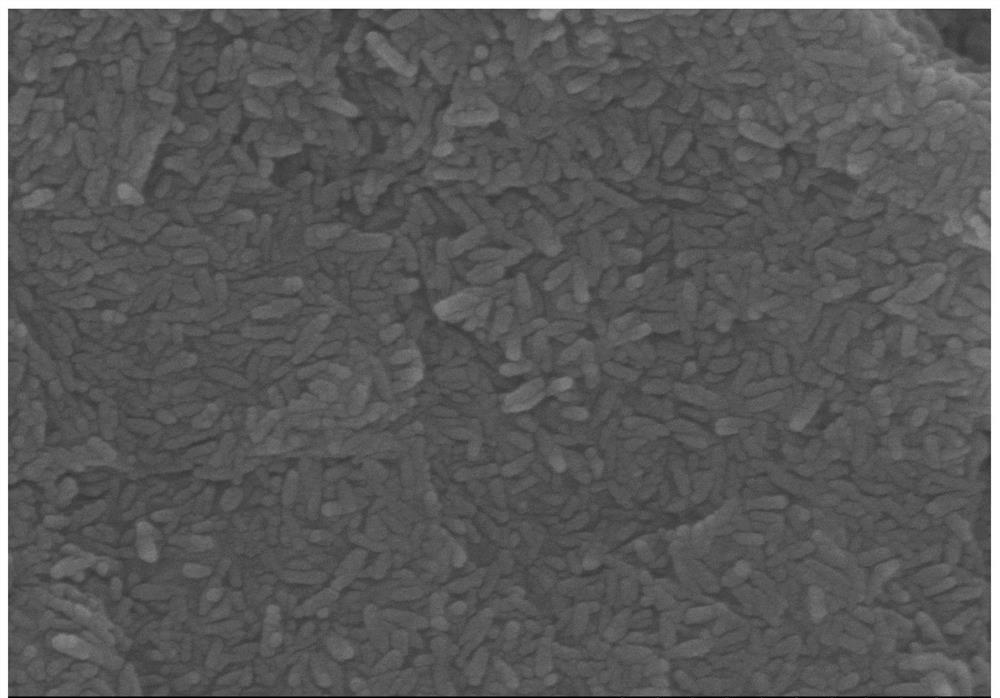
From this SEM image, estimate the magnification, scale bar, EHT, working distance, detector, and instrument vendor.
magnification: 90 K X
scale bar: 500 nm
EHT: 5 kV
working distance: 7 mm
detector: SE(M)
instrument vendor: Hitachi